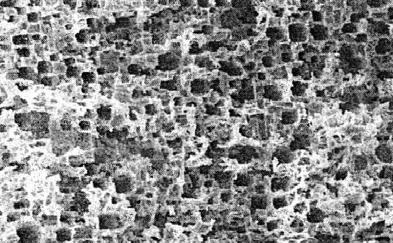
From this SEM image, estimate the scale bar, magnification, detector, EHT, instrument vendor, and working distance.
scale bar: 10000 nm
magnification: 1 K X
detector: SEI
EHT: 10 kV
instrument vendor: JEOL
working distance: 10.1 mm